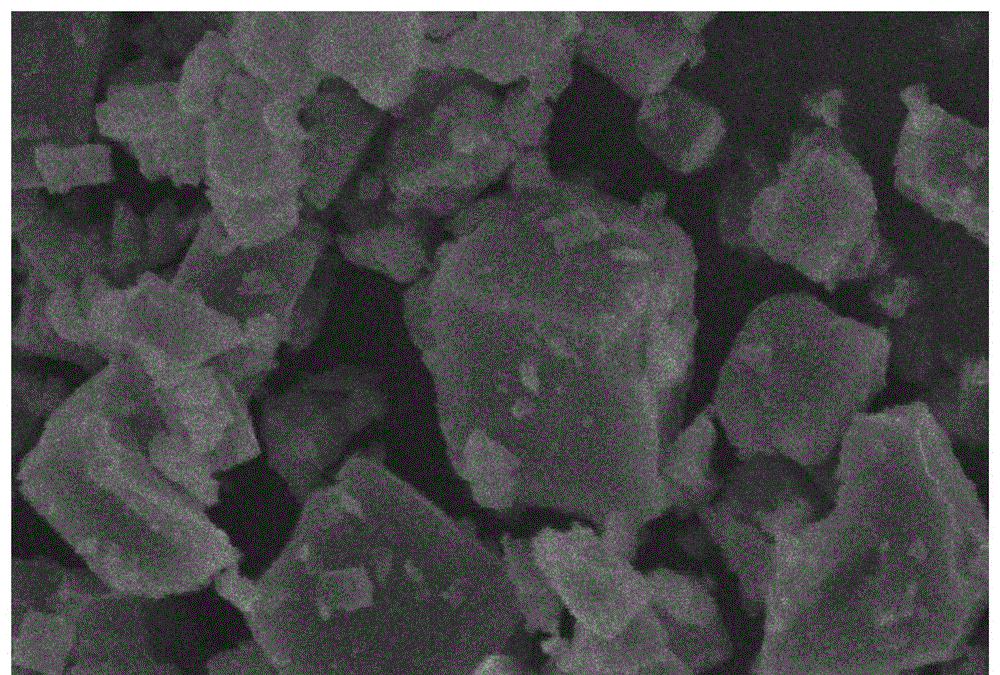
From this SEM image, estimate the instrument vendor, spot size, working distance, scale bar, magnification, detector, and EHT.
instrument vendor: other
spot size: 3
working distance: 7.2 mm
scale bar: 5000 nm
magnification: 10 K X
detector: SE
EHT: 13 kV